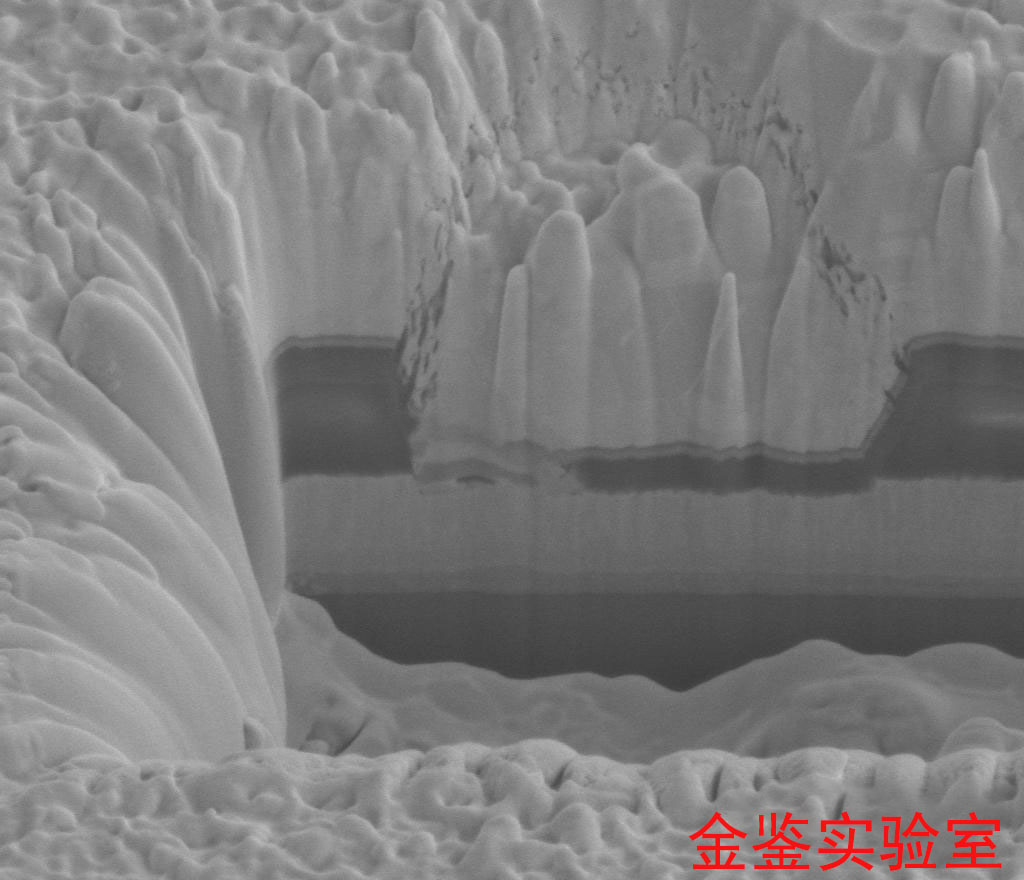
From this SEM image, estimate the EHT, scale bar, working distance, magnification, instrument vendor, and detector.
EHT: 5 kV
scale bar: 2000 nm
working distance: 4.9 mm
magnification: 40 K X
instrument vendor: FEI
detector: ETD